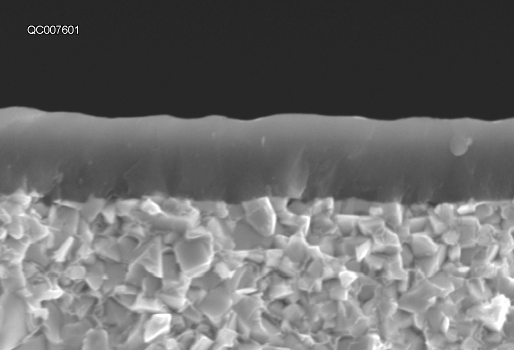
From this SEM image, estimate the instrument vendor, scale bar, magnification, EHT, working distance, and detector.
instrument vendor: Hitachi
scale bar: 5000 nm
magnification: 10 K X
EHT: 15 kV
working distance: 8.4 mm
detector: SE